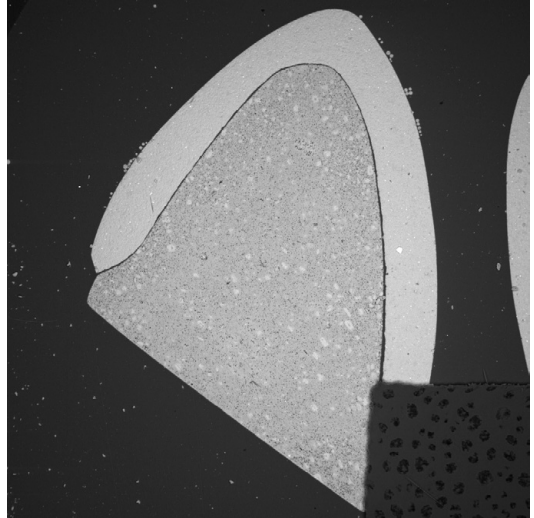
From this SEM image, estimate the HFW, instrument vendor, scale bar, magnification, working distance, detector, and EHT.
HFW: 8950 µm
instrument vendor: Tescan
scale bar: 2000000 nm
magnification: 0.028 K X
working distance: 14.94 mm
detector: BSE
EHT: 30 kV